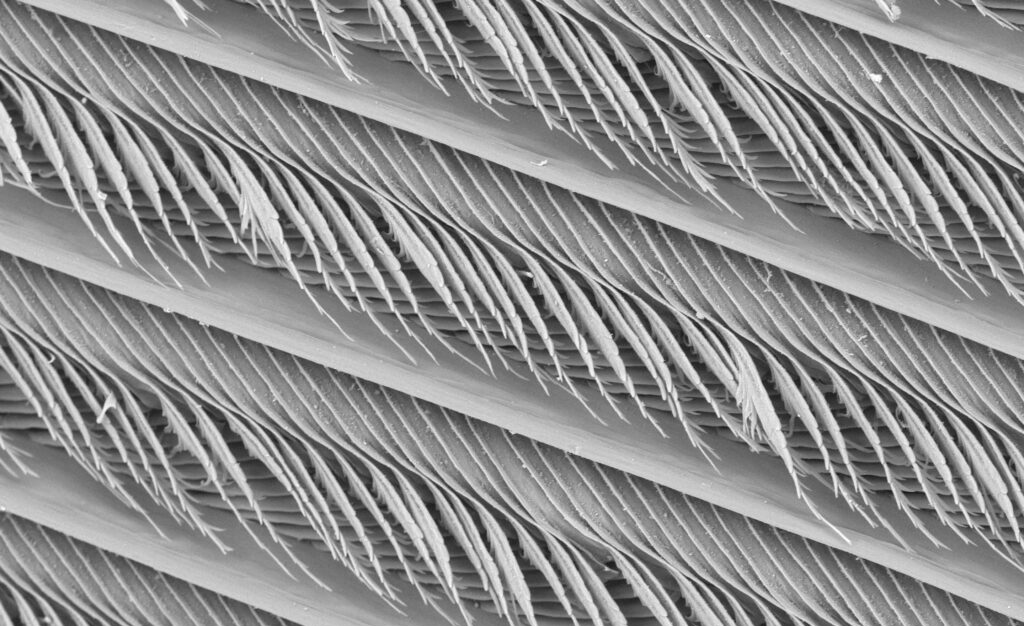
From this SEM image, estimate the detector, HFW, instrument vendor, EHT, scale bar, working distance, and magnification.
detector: BSD Full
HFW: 884 µm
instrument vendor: Thermo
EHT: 15 kV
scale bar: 200000 nm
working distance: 6.526 mm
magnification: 0.71 K X